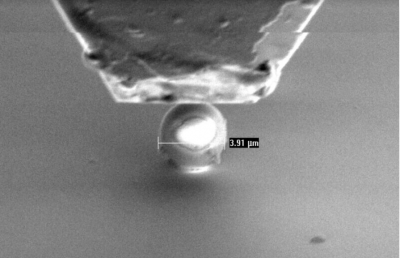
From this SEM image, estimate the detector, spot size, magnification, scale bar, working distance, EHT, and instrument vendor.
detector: SE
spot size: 3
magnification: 5 K X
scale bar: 5000 nm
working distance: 10 mm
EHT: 3 kV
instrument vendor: FEI